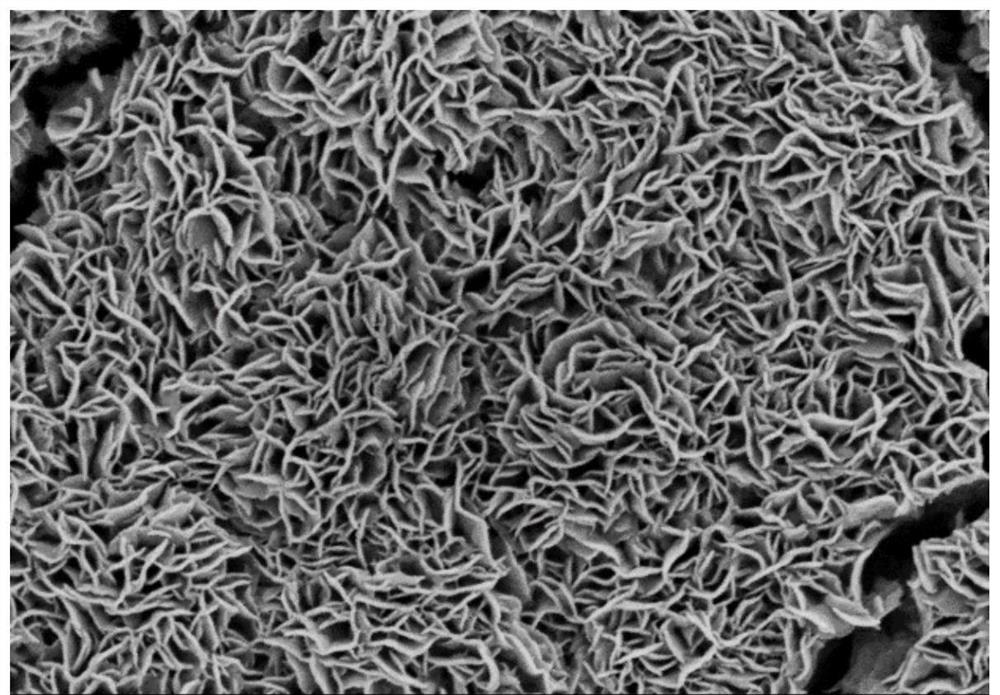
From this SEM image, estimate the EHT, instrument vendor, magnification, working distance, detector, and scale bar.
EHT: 2 kV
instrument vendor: Hitachi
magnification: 35 K X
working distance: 3.2 mm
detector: SE(M)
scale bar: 1000 nm